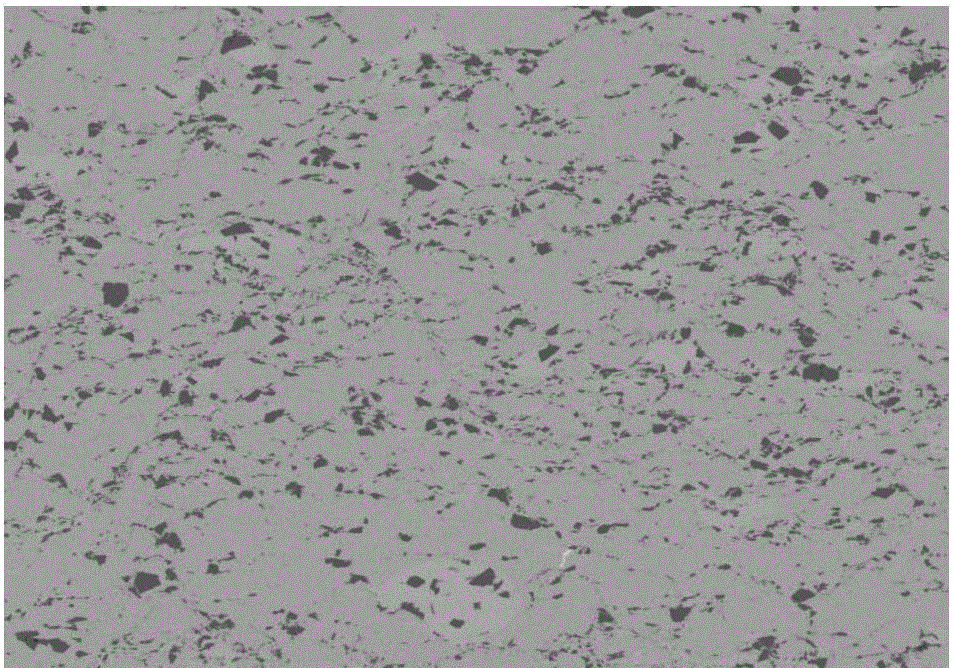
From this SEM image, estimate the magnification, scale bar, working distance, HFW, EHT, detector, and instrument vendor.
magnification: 0.2 K X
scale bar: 300000 nm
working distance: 13.1 mm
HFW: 635 µm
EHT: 20 kV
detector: vCD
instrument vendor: FEI